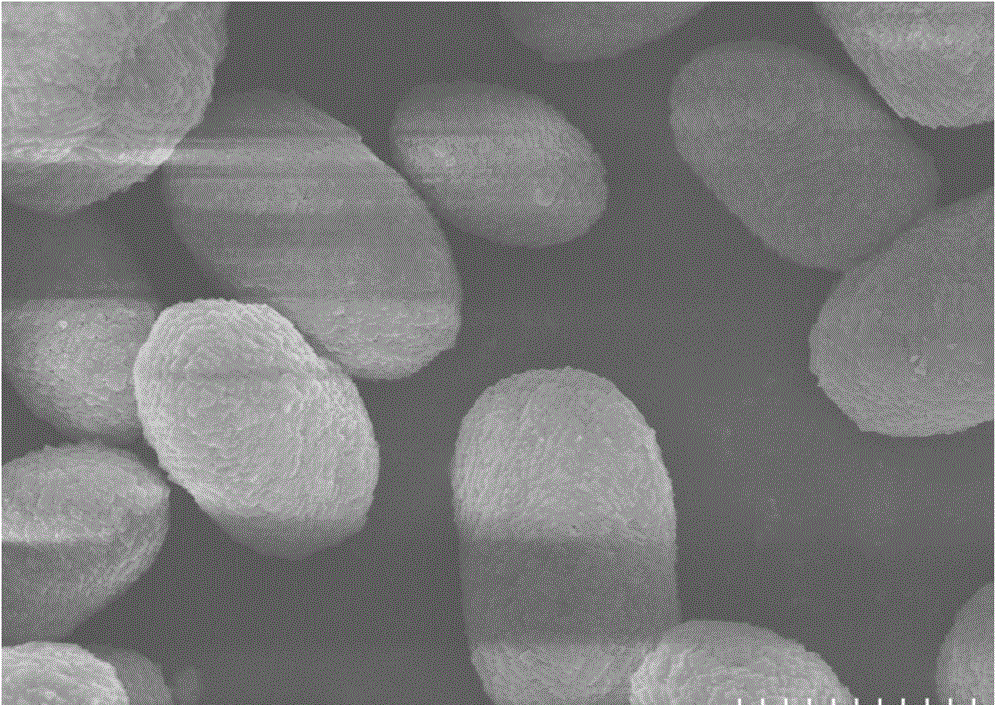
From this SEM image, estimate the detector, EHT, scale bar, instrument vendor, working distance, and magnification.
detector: SE(M)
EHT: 10 kV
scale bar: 5000 nm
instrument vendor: Hitachi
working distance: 8.4 mm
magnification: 6 K X